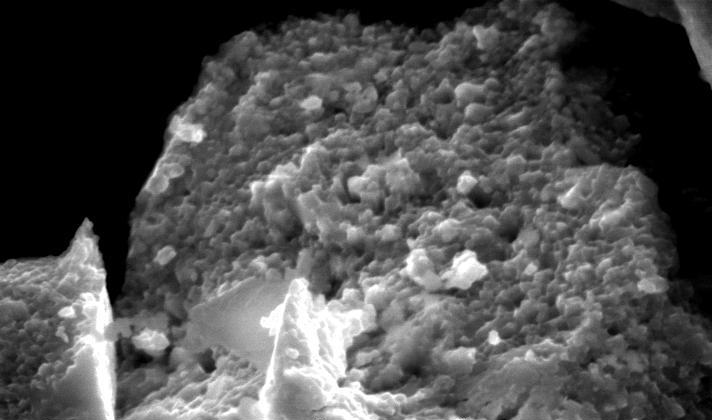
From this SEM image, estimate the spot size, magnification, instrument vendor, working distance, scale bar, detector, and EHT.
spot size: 3.5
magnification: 5 K X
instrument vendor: FEI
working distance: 13 mm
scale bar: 5000 nm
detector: SE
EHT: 30 kV